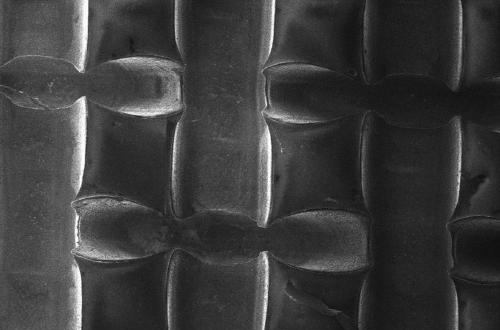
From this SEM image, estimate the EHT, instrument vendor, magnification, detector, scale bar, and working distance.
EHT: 2 kV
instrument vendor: Zeiss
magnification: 0.121 K X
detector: SE1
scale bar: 100000 nm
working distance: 13.5 mm